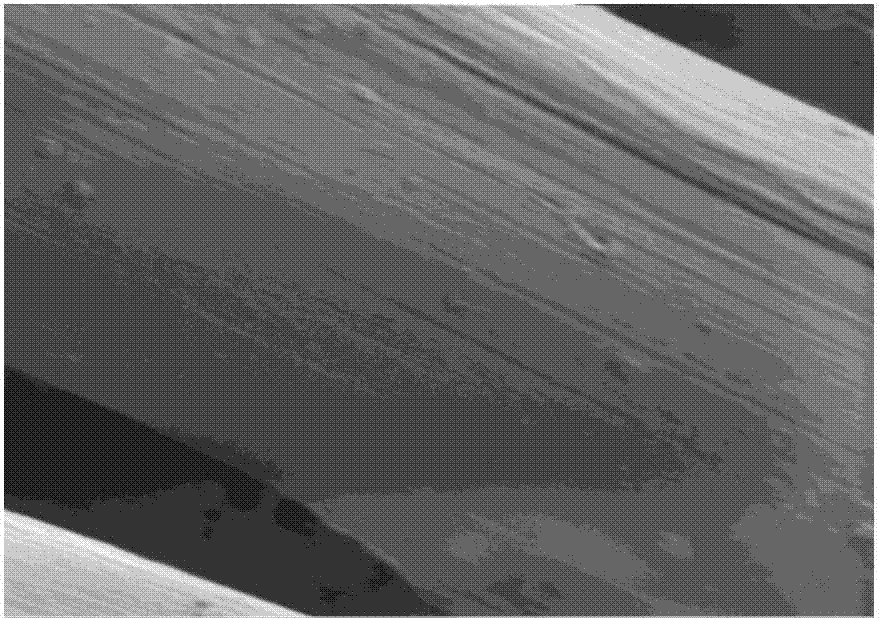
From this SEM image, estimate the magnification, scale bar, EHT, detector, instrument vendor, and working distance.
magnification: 5 K X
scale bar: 1000 nm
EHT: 1 kV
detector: SE2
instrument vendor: Zeiss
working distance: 5.7 mm